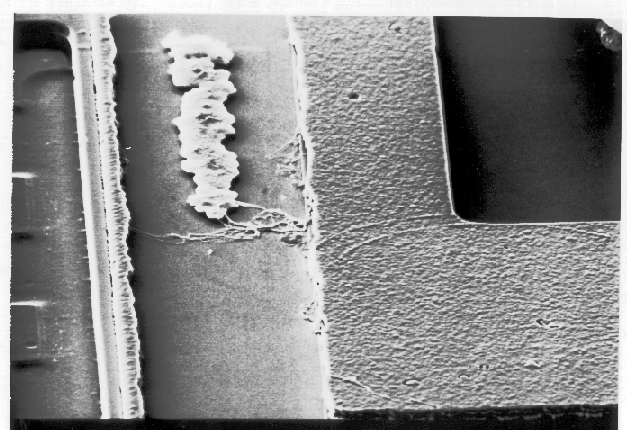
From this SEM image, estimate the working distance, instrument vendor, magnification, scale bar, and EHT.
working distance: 20 mm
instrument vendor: JEOL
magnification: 2.7 K X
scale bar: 10000 nm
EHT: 5 kV